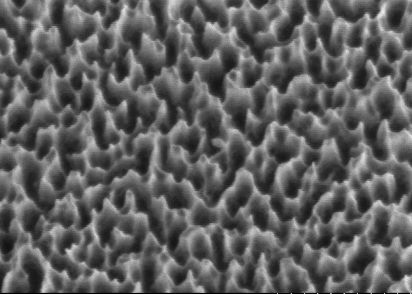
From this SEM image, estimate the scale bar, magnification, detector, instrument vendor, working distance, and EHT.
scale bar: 1000 nm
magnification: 40 K X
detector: SE(U)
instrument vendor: Hitachi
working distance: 8.8 mm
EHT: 1 kV